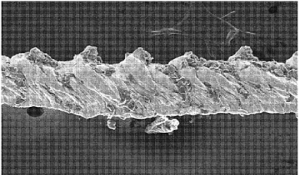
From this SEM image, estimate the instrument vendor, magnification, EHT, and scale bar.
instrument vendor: JEOL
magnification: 0.15 K X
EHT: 15 kV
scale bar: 100000 nm